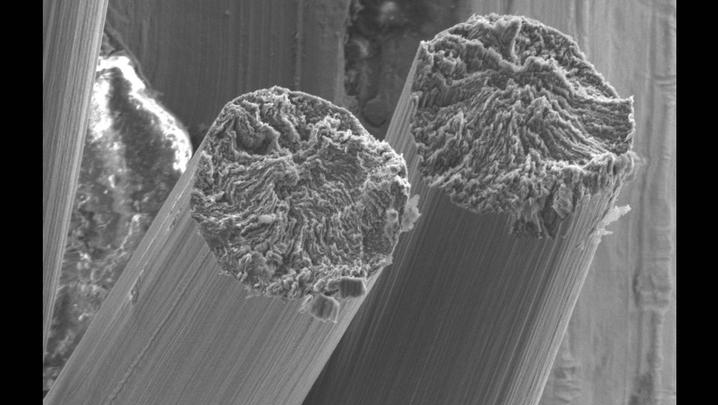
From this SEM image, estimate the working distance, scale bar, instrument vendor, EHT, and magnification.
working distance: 9 mm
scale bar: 20000 nm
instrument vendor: Hitachi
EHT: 5 kV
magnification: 2 K X